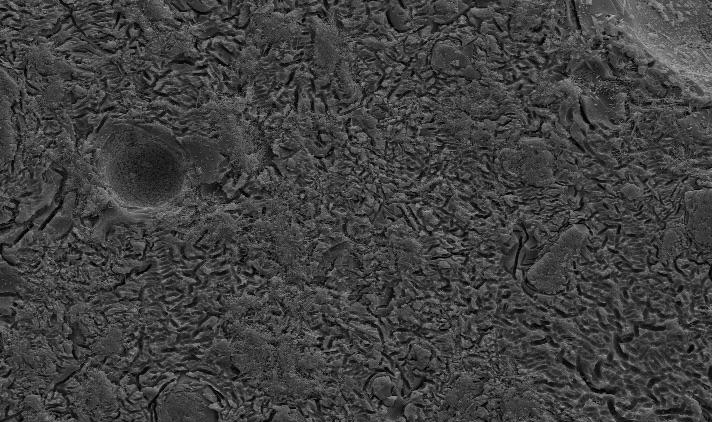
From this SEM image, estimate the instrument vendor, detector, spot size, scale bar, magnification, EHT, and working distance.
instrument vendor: FEI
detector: SE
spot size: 3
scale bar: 20000 nm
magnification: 1 K X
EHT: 15 kV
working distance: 7 mm